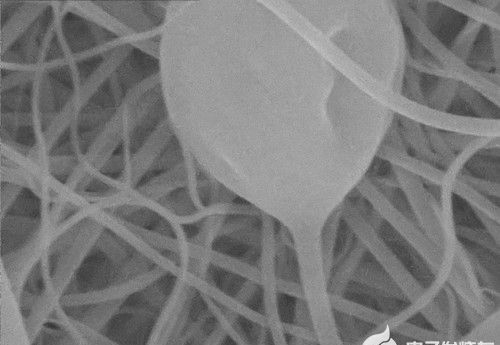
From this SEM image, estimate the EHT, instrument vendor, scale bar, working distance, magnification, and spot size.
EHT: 20 kV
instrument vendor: other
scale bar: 1000 nm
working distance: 5.9 mm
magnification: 50 K X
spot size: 5